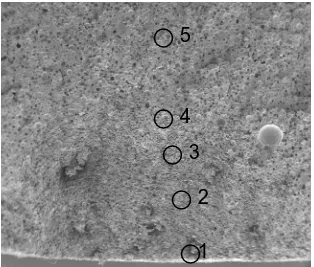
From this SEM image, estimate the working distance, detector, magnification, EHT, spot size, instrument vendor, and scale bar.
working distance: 28.6 mm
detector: ETD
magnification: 0.025 K X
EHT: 10 kV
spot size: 3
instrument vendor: FEI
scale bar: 2000000 nm